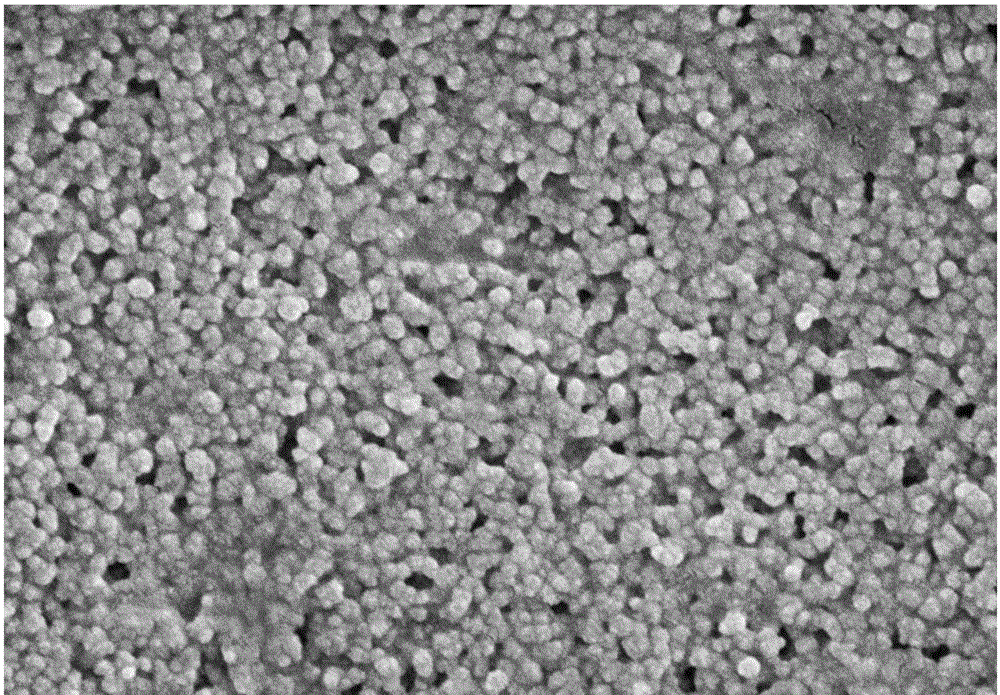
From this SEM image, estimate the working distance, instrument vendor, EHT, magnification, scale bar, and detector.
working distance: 9.1 mm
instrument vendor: Zeiss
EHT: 5 kV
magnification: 60 K X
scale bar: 200 nm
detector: SE2